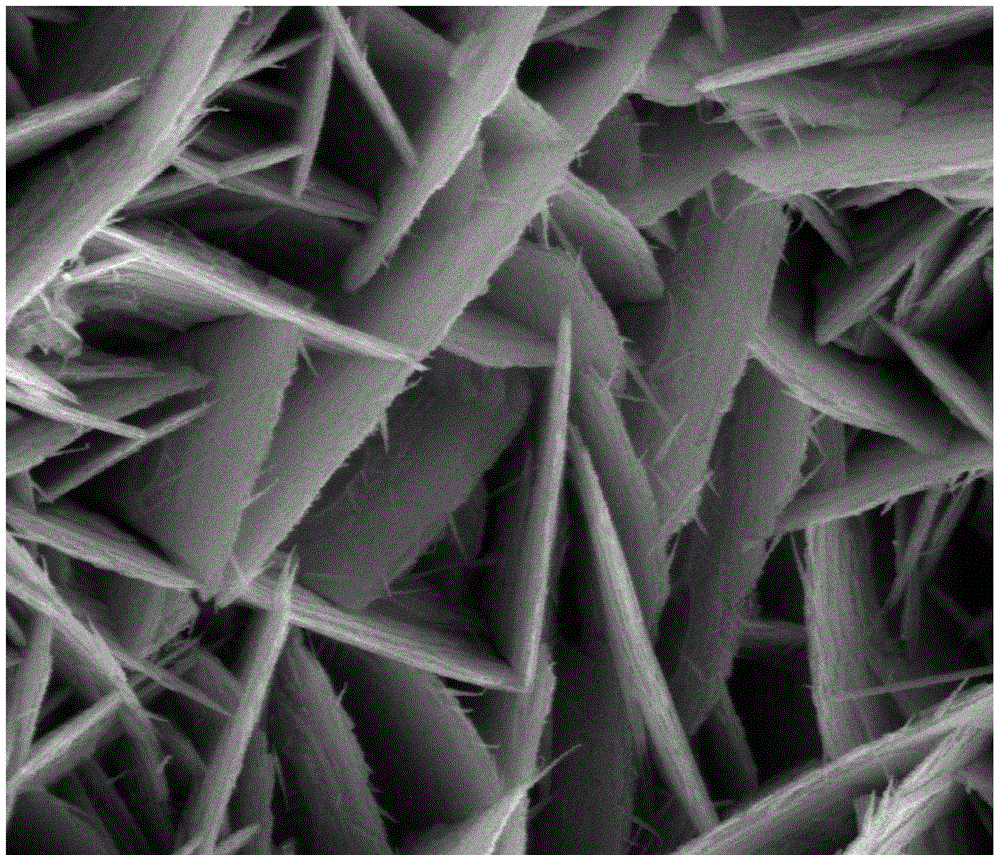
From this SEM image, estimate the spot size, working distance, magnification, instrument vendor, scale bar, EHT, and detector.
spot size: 2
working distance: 7.5 mm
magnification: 40 K X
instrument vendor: FEI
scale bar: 2000 nm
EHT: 10 kV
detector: ETD